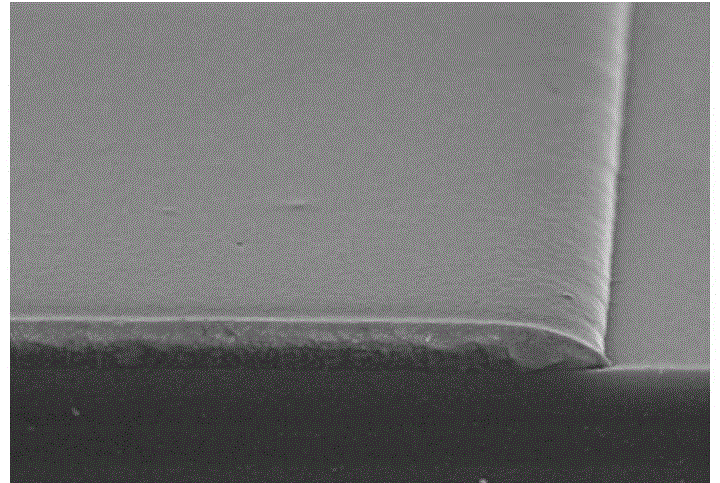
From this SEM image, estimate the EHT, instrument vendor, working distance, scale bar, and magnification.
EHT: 15 kV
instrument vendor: Hitachi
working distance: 11.8 mm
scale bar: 10000 nm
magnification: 5 K X